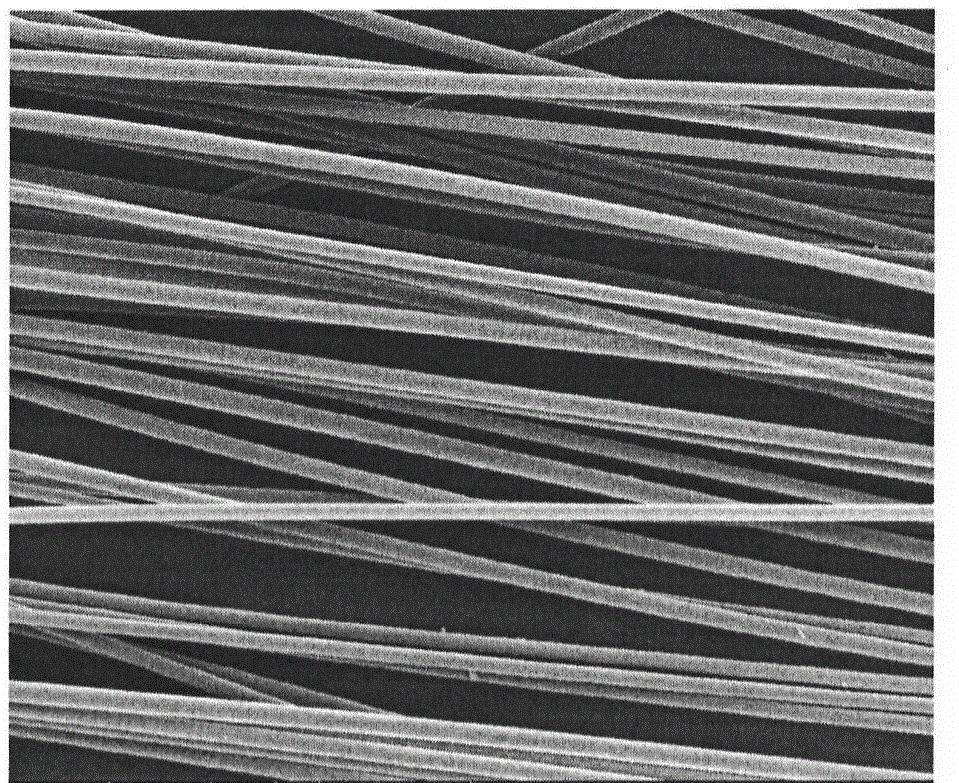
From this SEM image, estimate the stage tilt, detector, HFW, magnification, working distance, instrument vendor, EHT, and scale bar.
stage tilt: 0°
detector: ETD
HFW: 597 µm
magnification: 0.5 K X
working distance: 16.4 mm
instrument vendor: FEI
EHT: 15 kV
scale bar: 200000 nm